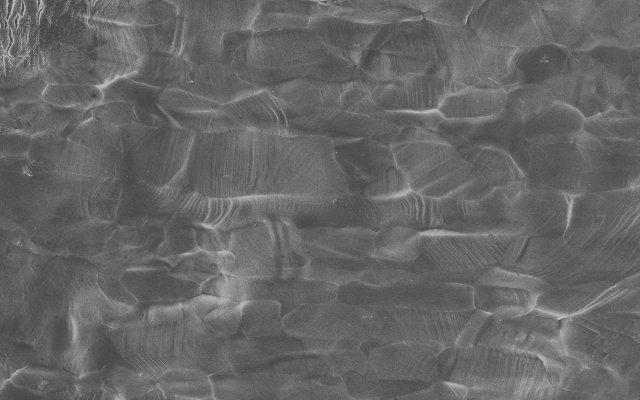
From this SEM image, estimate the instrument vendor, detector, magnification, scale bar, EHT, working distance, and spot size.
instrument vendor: FEI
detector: SE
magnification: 1 K X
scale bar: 10000 nm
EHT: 20 kV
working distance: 18 mm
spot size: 4